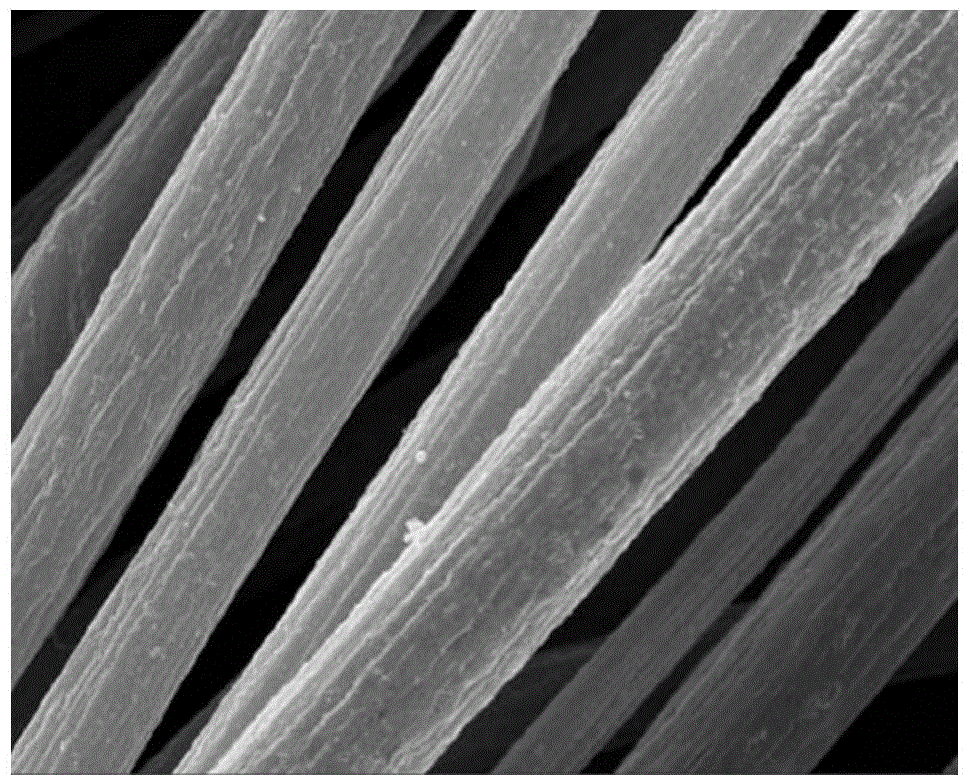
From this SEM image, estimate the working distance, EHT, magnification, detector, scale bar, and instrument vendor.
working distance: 7 mm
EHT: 10 kV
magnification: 10 K X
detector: InLens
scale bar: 1000 nm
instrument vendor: Zeiss